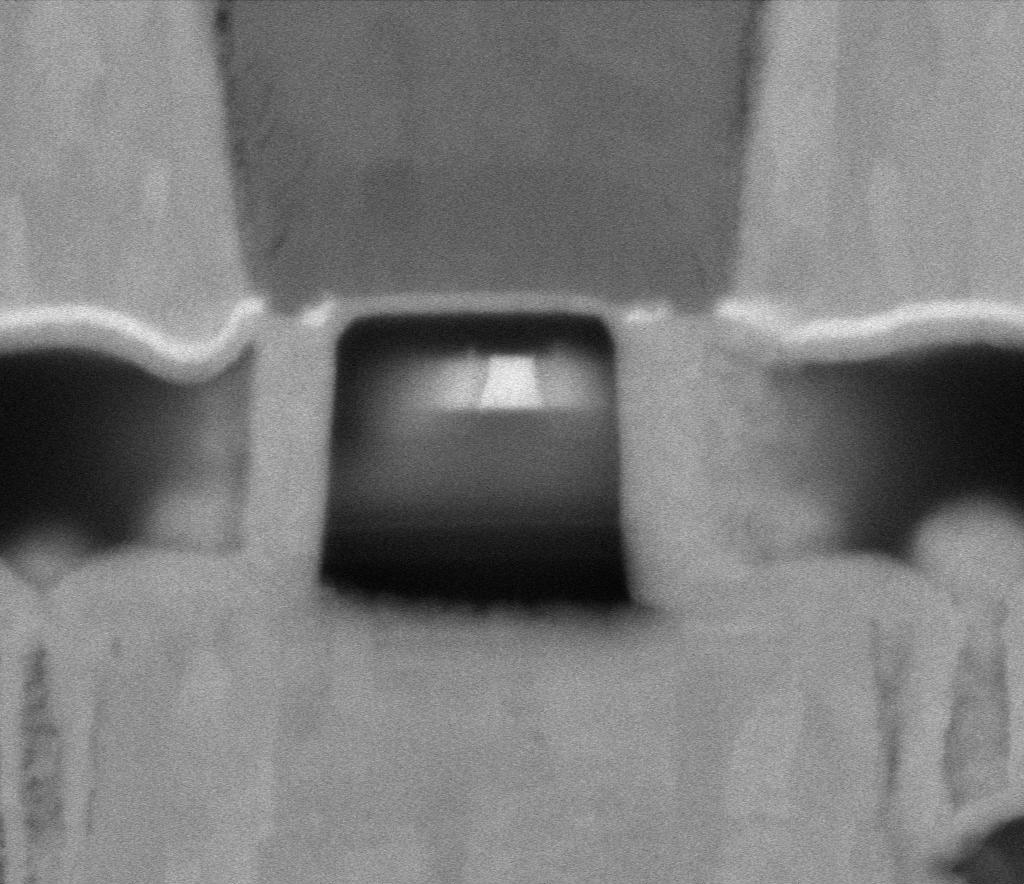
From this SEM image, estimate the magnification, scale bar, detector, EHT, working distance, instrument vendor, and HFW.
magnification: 568.609 K X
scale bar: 100 nm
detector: MD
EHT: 5 kV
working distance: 2 mm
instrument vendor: Thermo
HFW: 0.635 µm